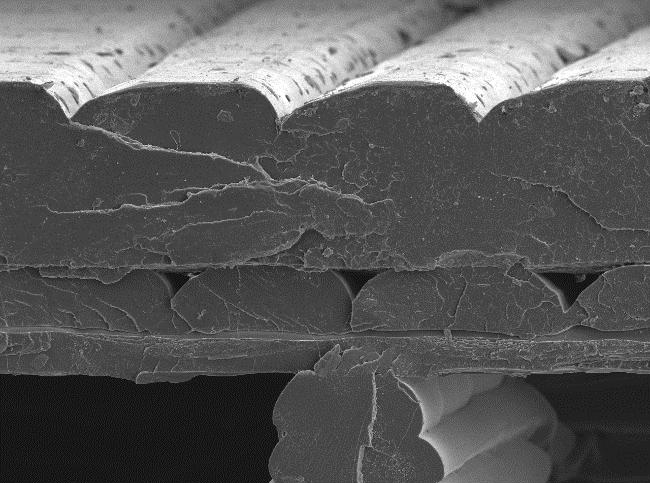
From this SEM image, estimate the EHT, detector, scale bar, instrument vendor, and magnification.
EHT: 15 kV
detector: SEI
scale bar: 100000 nm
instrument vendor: JEOL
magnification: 0.1 K X